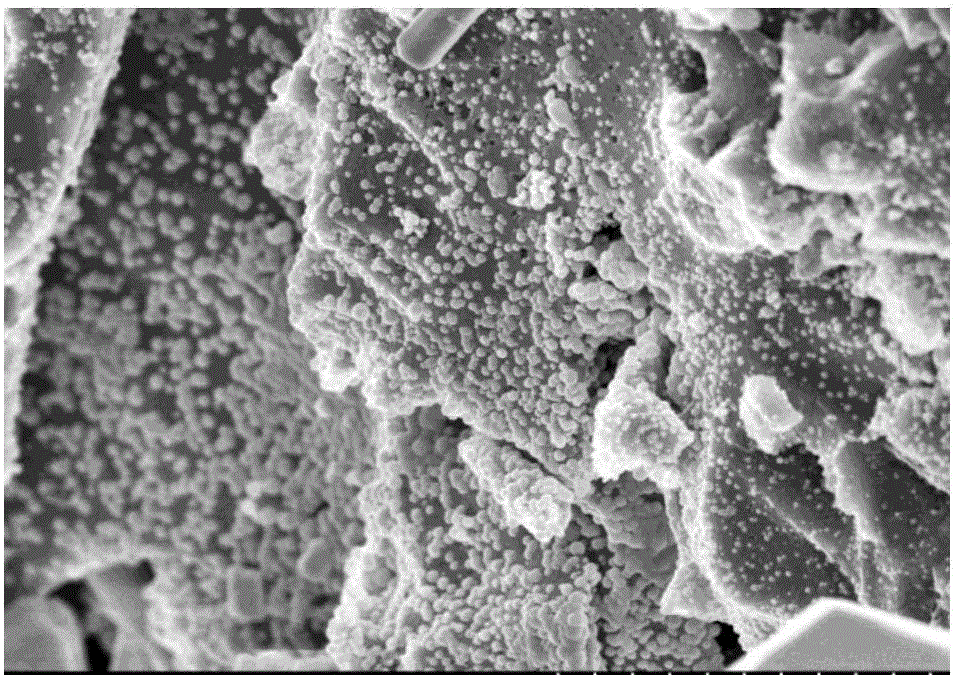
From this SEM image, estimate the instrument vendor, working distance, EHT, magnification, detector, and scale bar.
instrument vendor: Hitachi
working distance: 11.1 mm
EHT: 5 kV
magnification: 10 K X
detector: SE(U)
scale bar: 5000 nm